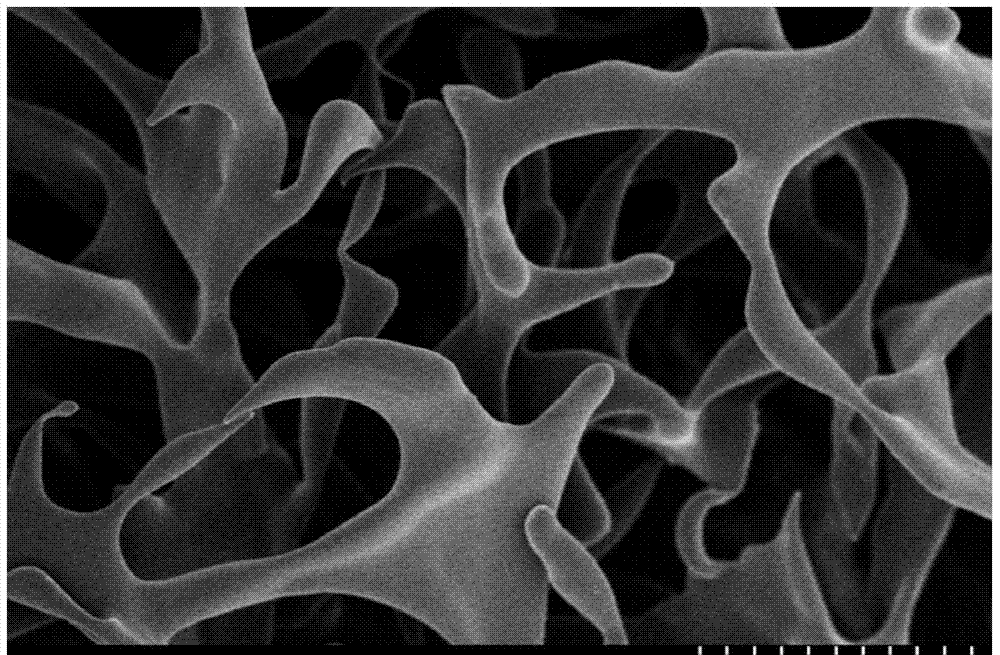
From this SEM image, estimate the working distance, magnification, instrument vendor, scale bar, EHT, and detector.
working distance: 7.1 mm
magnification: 3.5 K X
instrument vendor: Hitachi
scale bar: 10000 nm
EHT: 3 kV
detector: SE(M)